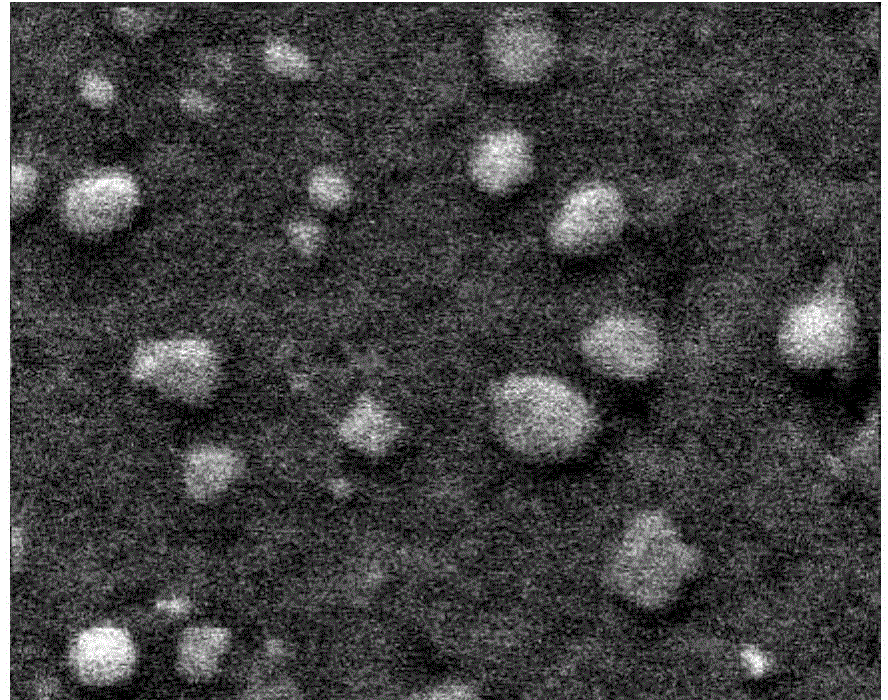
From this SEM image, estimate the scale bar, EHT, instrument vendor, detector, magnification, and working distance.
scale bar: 1000 nm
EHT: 10 kV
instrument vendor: Hitachi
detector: SE(UL)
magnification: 50 K X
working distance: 12.7 mm